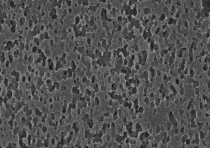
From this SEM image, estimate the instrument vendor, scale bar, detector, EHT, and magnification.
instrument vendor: JEOL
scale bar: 5000 nm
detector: SEI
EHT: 15 kV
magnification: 5 K X